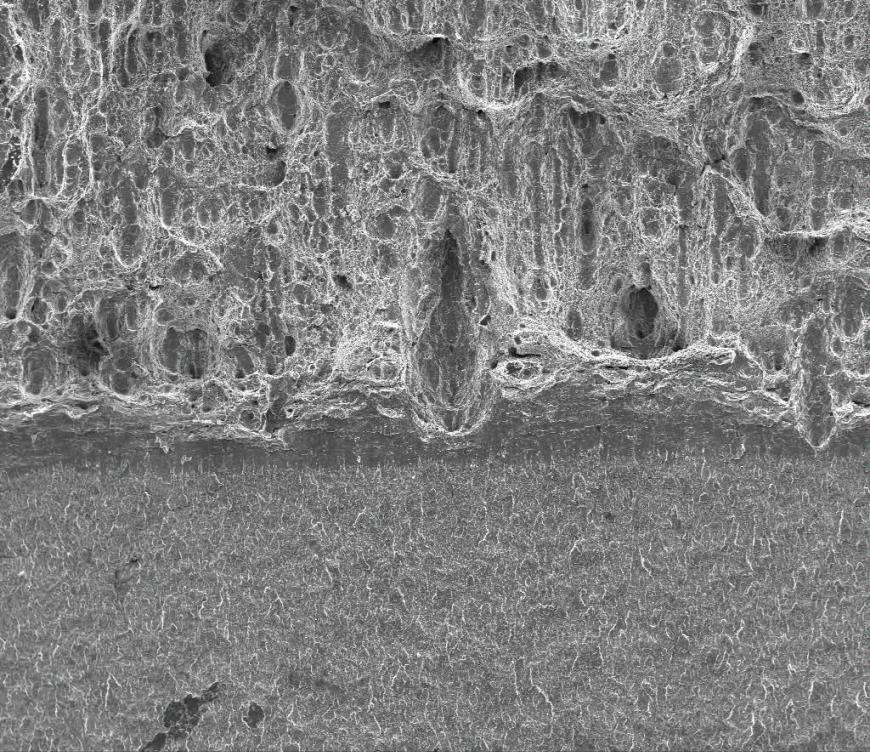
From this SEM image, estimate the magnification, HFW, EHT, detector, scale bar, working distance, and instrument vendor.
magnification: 0.1 K X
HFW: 2980 µm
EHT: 25 kV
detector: ETD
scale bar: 1000000 nm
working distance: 38.6 mm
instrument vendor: FEI